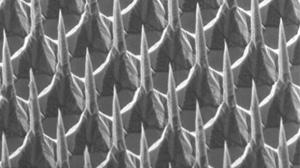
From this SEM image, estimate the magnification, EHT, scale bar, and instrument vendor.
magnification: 0.2 K X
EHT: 25 kV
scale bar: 200000 nm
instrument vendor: JEOL